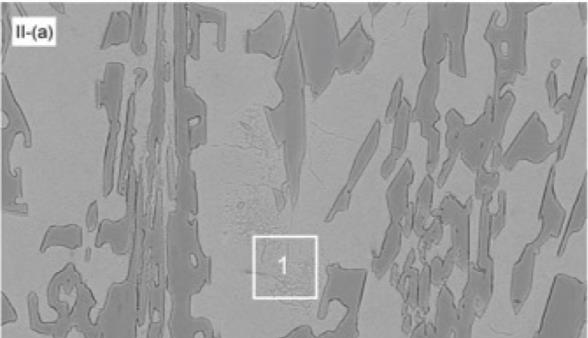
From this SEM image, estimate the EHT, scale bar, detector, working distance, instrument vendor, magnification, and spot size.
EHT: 20 kV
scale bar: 50000 nm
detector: BSE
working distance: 9.9 mm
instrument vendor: FEI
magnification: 0.5 K X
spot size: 5